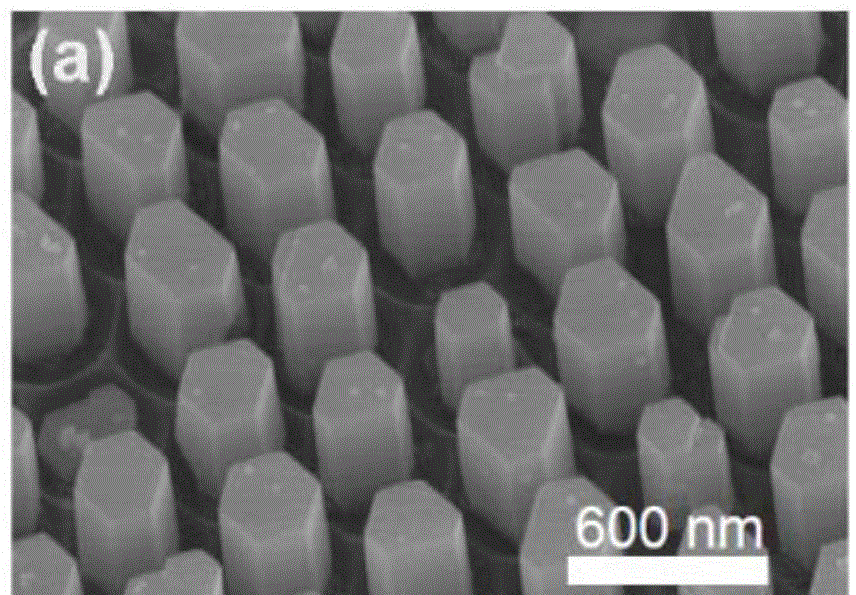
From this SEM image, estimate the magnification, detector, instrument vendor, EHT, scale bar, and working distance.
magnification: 50 K X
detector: SE(M)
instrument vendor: Hitachi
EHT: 10 kV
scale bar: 1000 nm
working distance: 8 mm